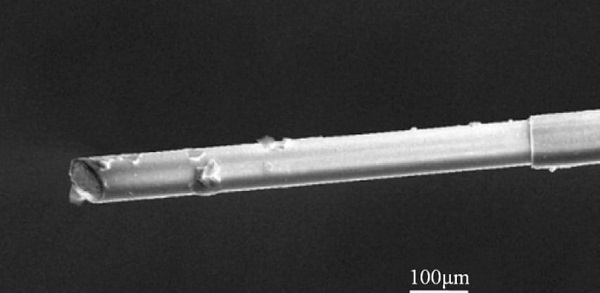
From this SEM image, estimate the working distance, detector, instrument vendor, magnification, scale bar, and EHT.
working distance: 14.2 mm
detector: SE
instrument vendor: FEI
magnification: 0.5 K X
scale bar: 100000 nm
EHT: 20 kV